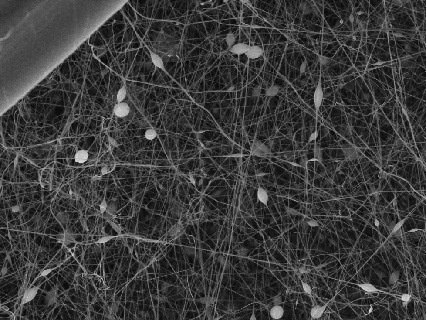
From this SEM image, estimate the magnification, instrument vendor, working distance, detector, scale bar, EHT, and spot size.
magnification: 2 K X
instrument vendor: other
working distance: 7.51 mm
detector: SEI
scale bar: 6000 nm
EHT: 20 kV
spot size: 1